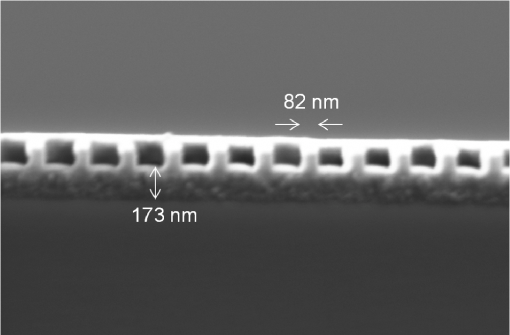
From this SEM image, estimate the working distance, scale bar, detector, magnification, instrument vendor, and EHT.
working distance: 15.2 mm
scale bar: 200 nm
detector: SE2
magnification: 37.78 K X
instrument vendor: Zeiss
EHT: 5 kV